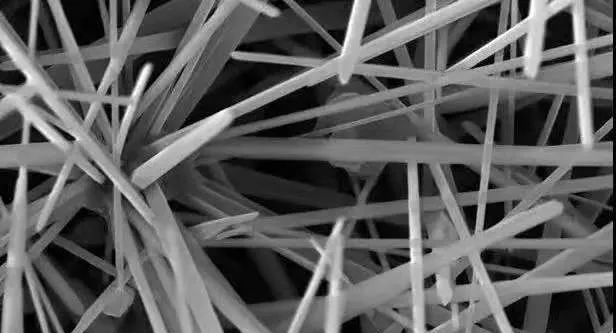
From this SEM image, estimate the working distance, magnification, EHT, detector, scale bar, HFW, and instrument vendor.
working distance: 4.99 mm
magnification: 20 K X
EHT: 10 kV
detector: SE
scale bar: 2000 nm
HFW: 10.4 µm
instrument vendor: Tescan